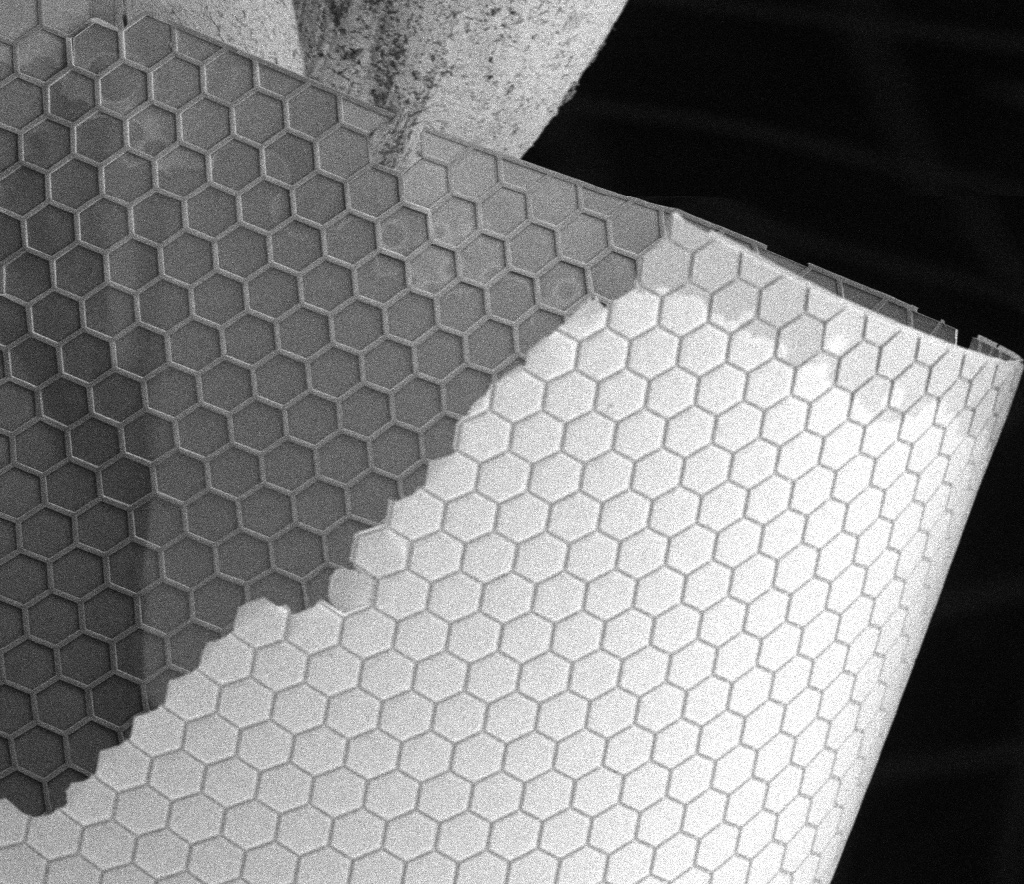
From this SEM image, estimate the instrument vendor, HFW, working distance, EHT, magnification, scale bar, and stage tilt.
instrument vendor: FEI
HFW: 1070 µm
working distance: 9.8 mm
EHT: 5 kV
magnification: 0.12 K X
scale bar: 400000 nm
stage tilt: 0°